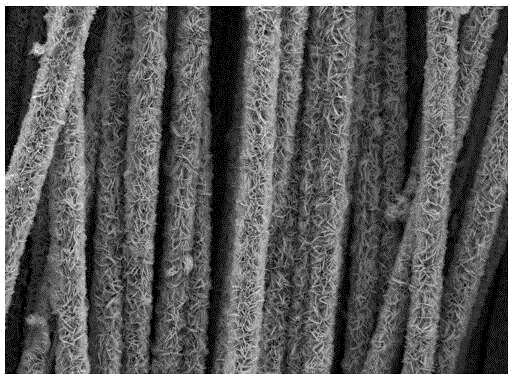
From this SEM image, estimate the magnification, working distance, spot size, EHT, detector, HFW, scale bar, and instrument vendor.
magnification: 2 K X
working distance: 10.7 mm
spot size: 3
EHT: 20 kV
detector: SE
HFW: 207 µm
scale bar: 50000 nm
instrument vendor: FEI